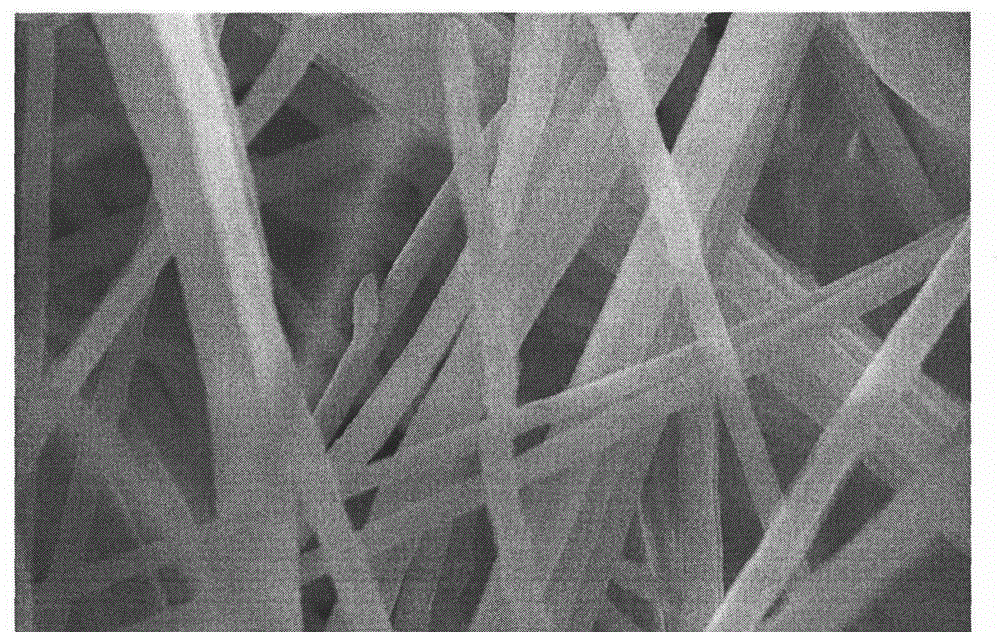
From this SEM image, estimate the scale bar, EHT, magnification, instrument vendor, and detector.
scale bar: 10000 nm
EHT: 20 kV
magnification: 3.5 K X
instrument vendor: Hitachi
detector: SE(M)